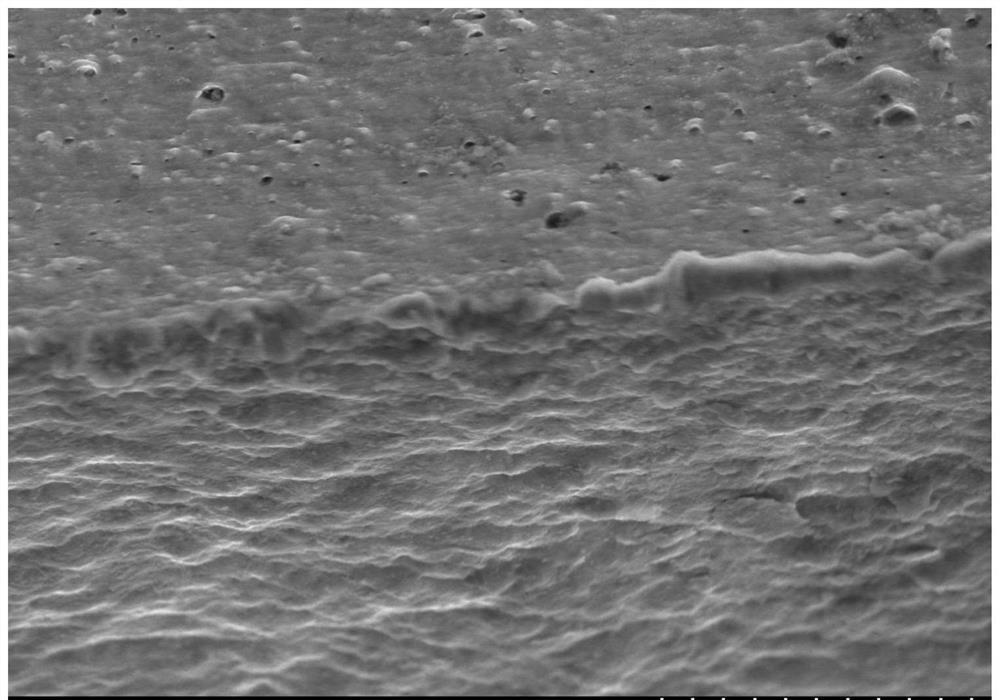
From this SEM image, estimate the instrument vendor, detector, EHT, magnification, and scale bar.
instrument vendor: Hitachi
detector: SE(M)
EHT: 10 kV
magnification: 2 K X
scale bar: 20000 nm